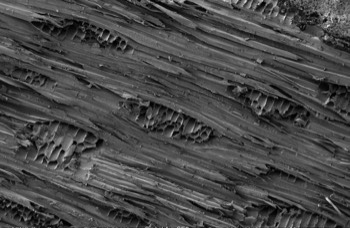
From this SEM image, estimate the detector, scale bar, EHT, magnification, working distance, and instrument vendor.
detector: SE2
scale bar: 100000 nm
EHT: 3 kV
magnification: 0.1 K X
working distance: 17.6 mm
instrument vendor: Zeiss